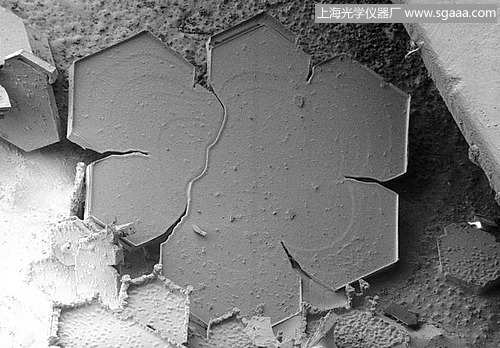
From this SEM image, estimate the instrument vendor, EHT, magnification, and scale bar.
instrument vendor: JEOL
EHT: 2 kV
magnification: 0.04 K X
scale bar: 750000 nm